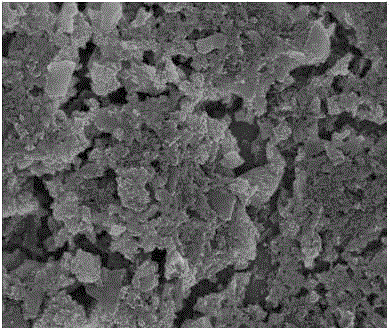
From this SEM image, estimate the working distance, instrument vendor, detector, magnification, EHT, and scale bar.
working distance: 5.7 mm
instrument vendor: FEI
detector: TLD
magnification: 25 K X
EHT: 5 kV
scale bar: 1000 nm